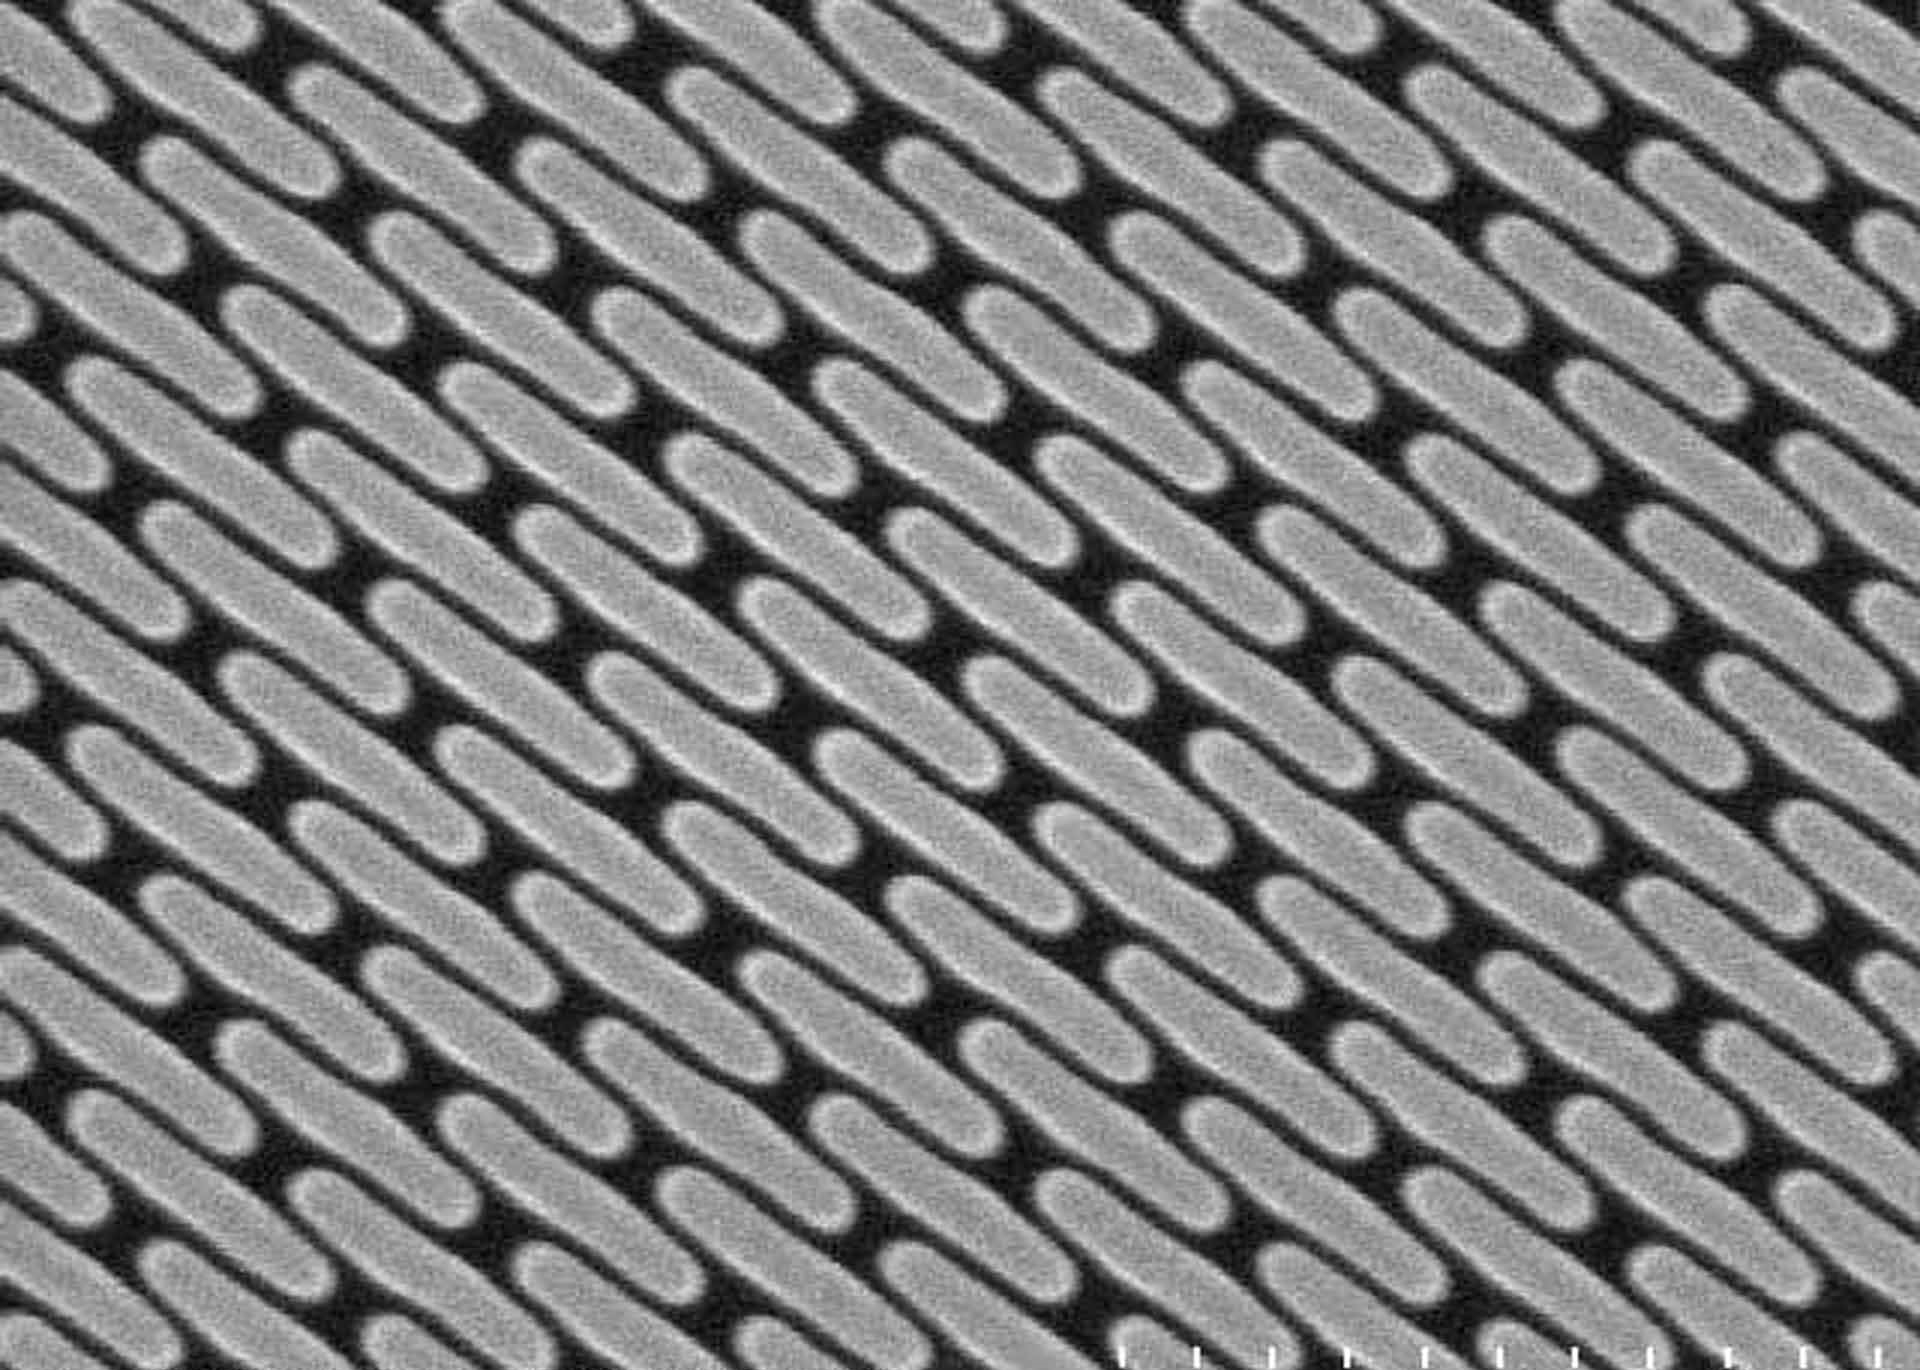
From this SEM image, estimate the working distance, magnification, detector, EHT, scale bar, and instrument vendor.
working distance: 18.5 mm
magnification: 100 K X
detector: SE(M)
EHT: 15 kV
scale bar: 500 nm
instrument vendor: Hitachi